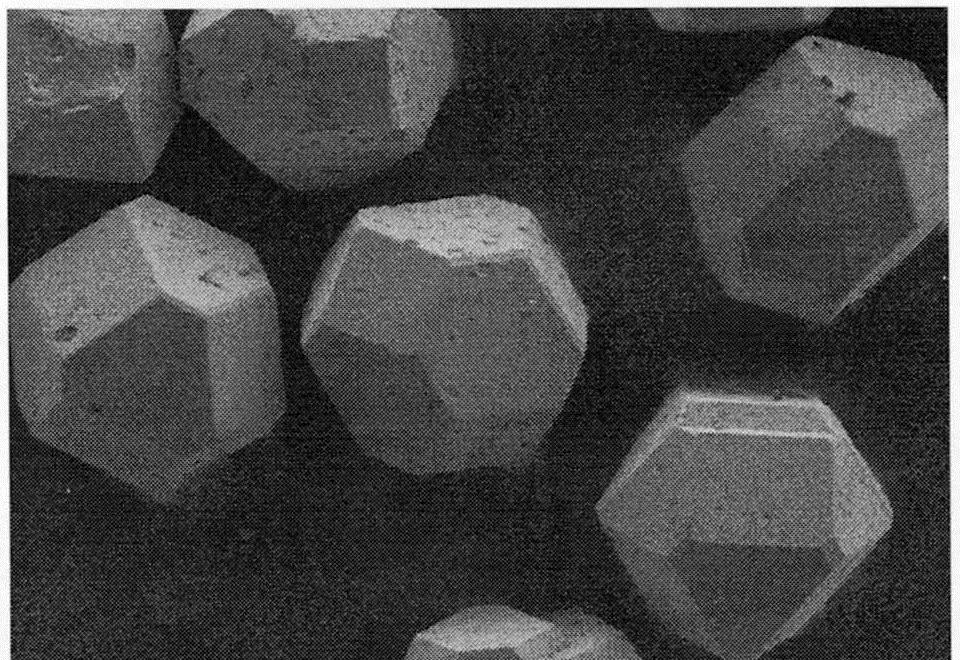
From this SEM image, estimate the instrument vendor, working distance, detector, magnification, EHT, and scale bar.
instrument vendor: Hitachi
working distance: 9.1 mm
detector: SE(M)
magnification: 0.2 K X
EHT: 5 kV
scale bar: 200000 nm